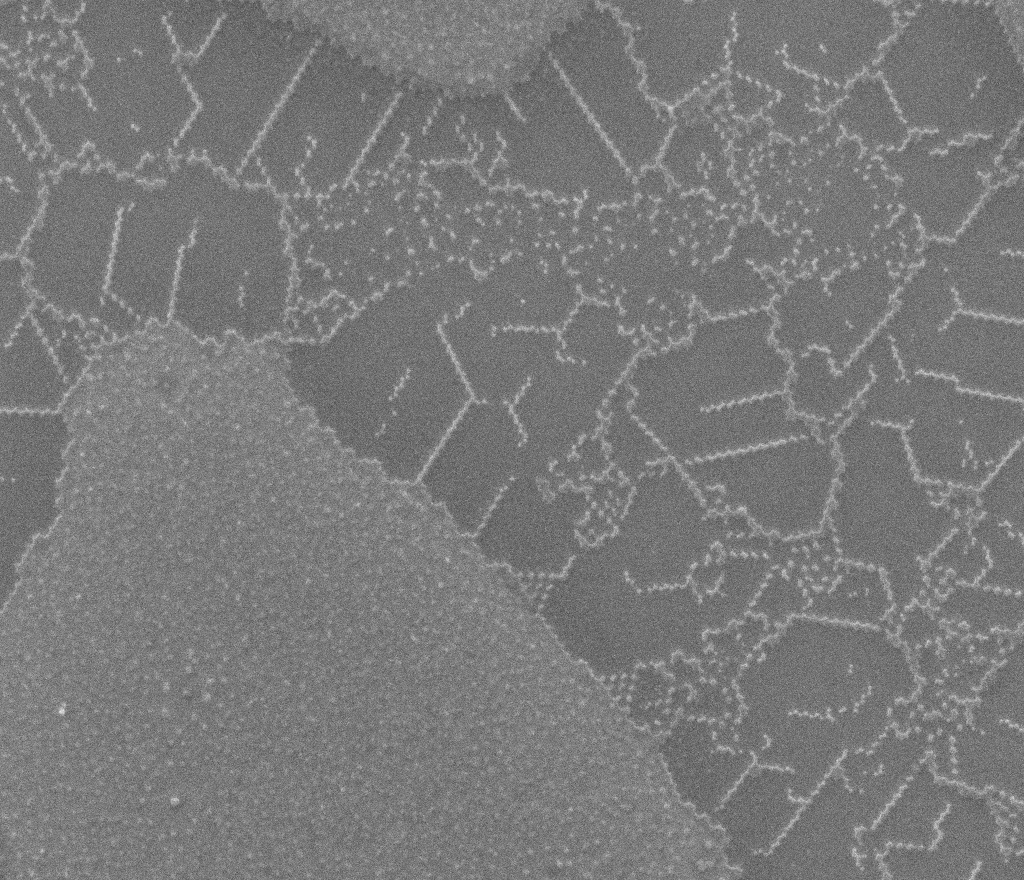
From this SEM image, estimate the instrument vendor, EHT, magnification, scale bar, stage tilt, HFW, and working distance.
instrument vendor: FEI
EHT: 25 kV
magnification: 10.758 K X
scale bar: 5000 nm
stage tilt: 0°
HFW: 25.1 µm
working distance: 12.3 mm